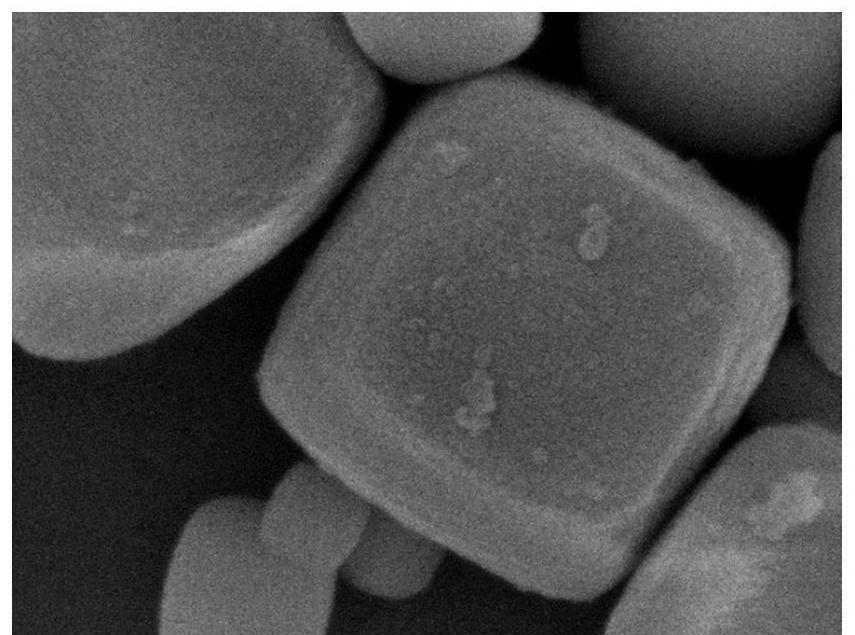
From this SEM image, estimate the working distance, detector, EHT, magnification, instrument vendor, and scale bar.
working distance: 6.4 mm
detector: SEI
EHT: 8 kV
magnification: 50 K X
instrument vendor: JEOL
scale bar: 100 nm